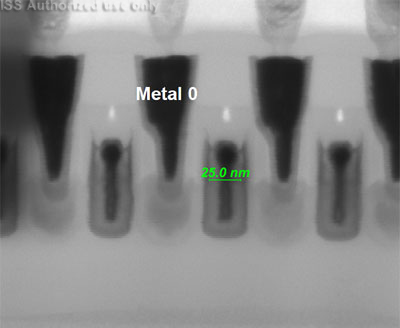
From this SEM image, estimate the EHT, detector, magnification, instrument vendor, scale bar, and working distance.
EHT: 30 kV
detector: STEM II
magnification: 800 K X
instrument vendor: FEI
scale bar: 100 nm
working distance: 4.1 mm